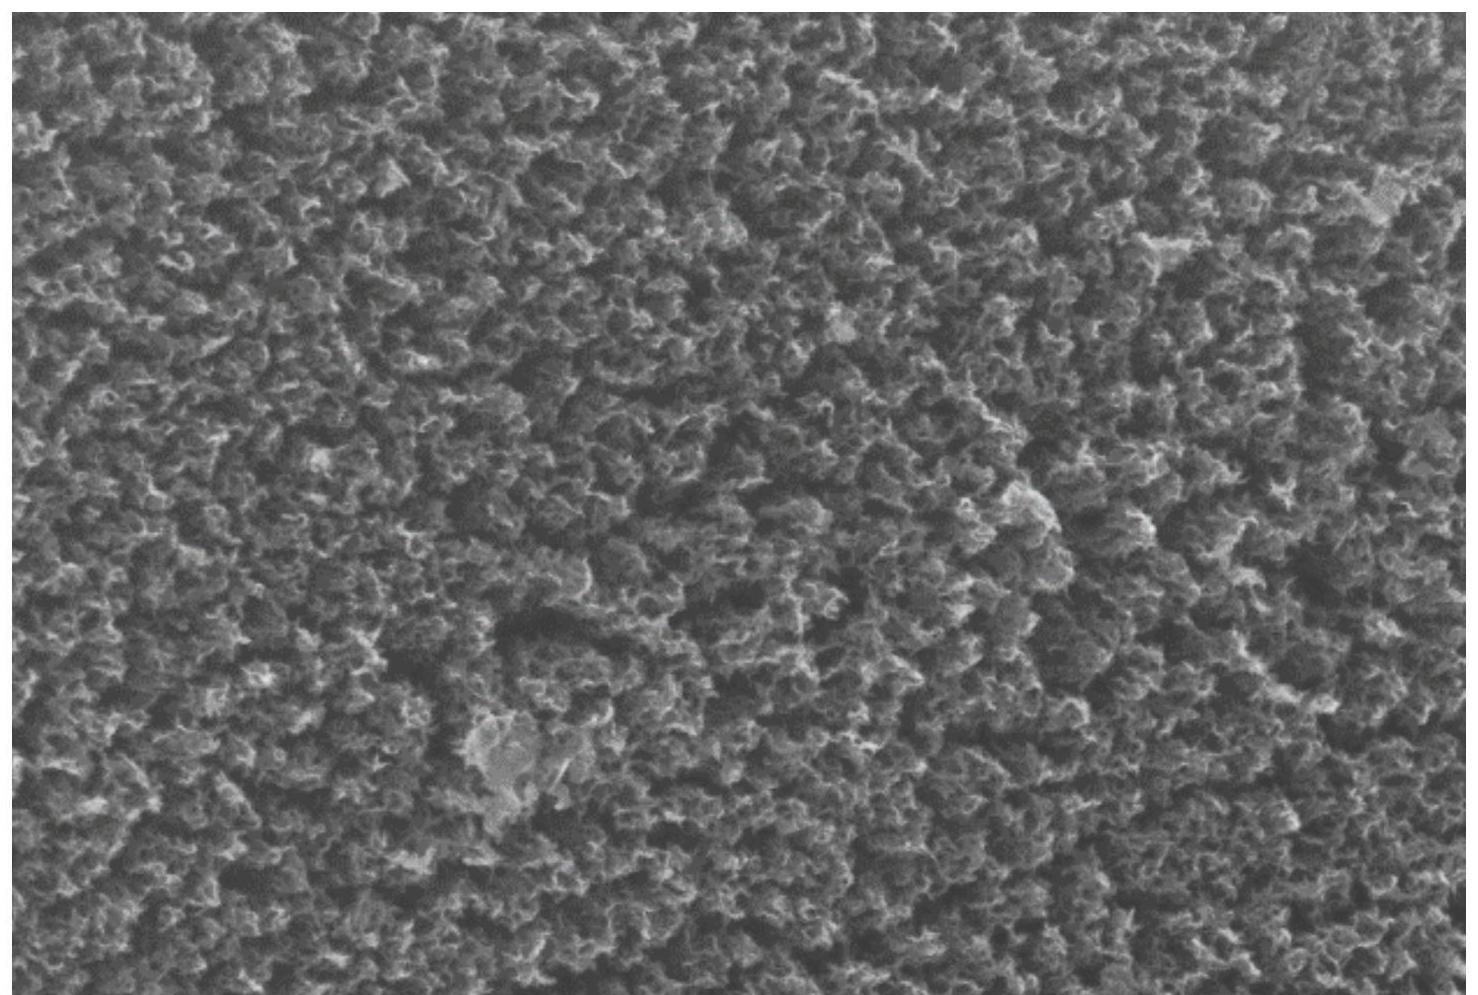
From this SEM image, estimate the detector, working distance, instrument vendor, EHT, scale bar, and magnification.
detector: InLens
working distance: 4.7 mm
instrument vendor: Zeiss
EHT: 5 kV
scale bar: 100 nm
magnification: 50 K X